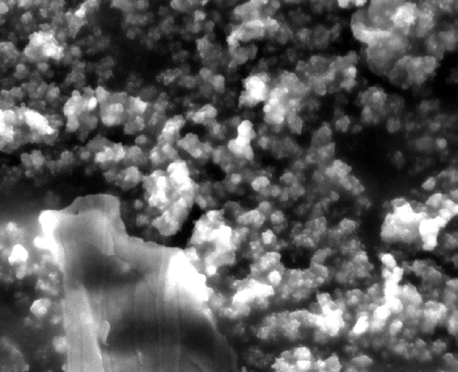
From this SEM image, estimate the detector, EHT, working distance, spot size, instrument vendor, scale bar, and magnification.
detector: ETD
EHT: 30 kV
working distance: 10.3 mm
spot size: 5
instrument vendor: FEI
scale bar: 20000 nm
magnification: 2 K X